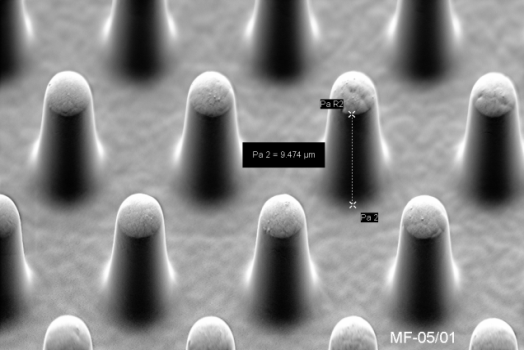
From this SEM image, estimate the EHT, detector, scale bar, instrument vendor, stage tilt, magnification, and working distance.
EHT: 2 kV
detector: SE2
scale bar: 2000 nm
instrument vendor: Zeiss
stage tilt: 45°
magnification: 5 K X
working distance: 6.6 mm